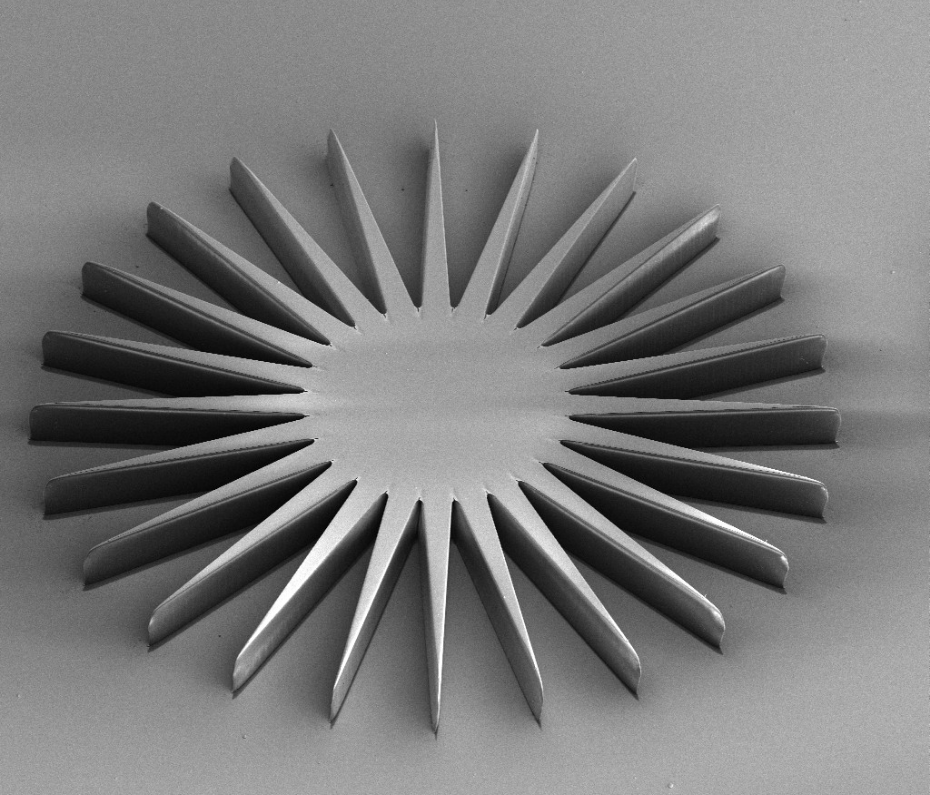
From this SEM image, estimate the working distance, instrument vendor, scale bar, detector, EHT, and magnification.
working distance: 41.3 mm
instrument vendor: FEI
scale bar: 400000 nm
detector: ETD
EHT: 5 kV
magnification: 0.141 K X